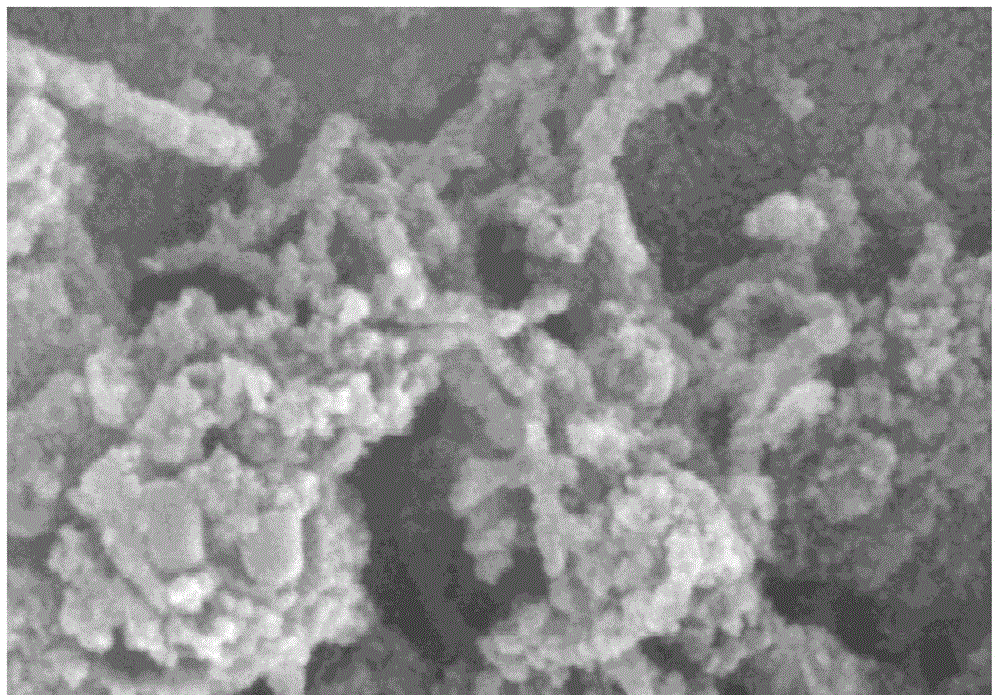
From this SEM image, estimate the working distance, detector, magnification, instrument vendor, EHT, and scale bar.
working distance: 15.5 mm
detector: SE(M)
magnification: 100 K X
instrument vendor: Hitachi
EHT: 15 kV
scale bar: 500 nm